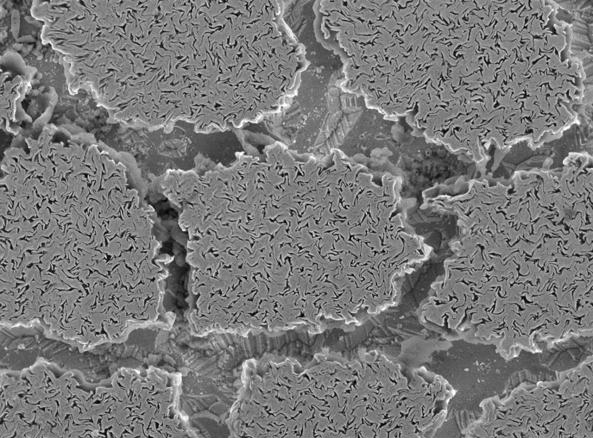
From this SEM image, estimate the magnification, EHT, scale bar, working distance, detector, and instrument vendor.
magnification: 5.5 K X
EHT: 5 kV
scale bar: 1000 nm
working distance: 8 mm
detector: SEI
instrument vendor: JEOL